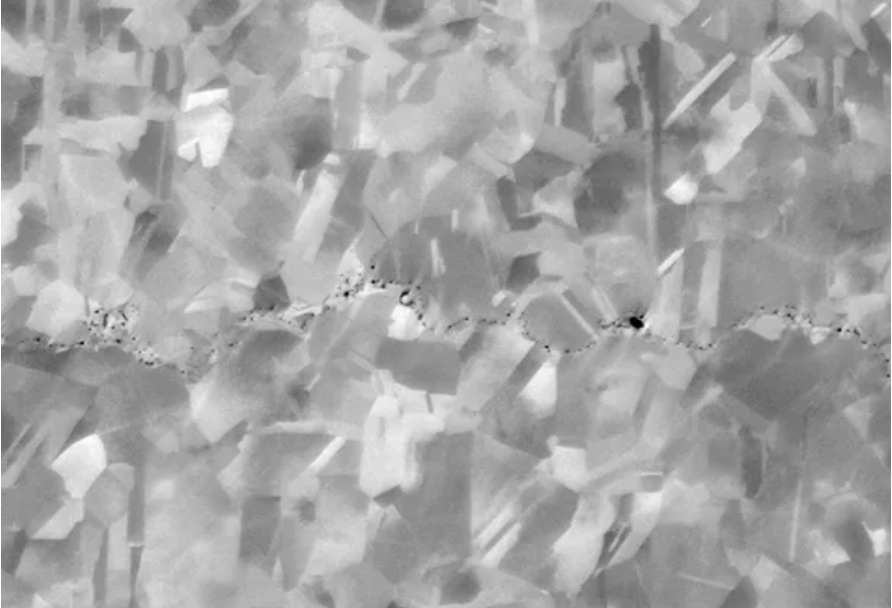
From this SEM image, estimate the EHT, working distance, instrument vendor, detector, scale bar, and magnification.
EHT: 8 kV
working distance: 6.5 mm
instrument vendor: Zeiss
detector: NTS BSD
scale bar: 1000 nm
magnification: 8 K X